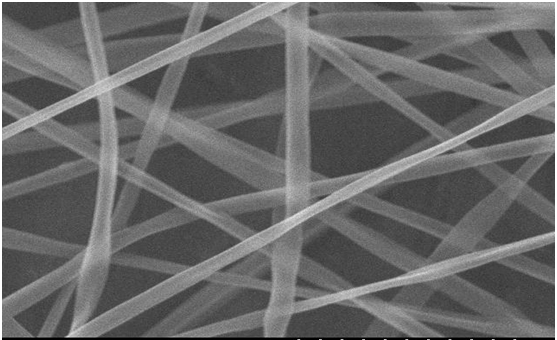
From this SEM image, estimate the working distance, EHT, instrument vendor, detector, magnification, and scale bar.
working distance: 9.4 mm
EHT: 20 kV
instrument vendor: Hitachi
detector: SE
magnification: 10 K X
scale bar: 5000 nm